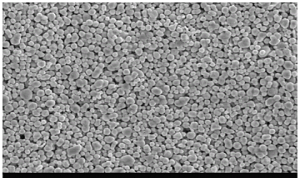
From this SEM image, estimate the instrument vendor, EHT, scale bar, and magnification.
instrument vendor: other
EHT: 20 kV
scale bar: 10000 nm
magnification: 2 K X